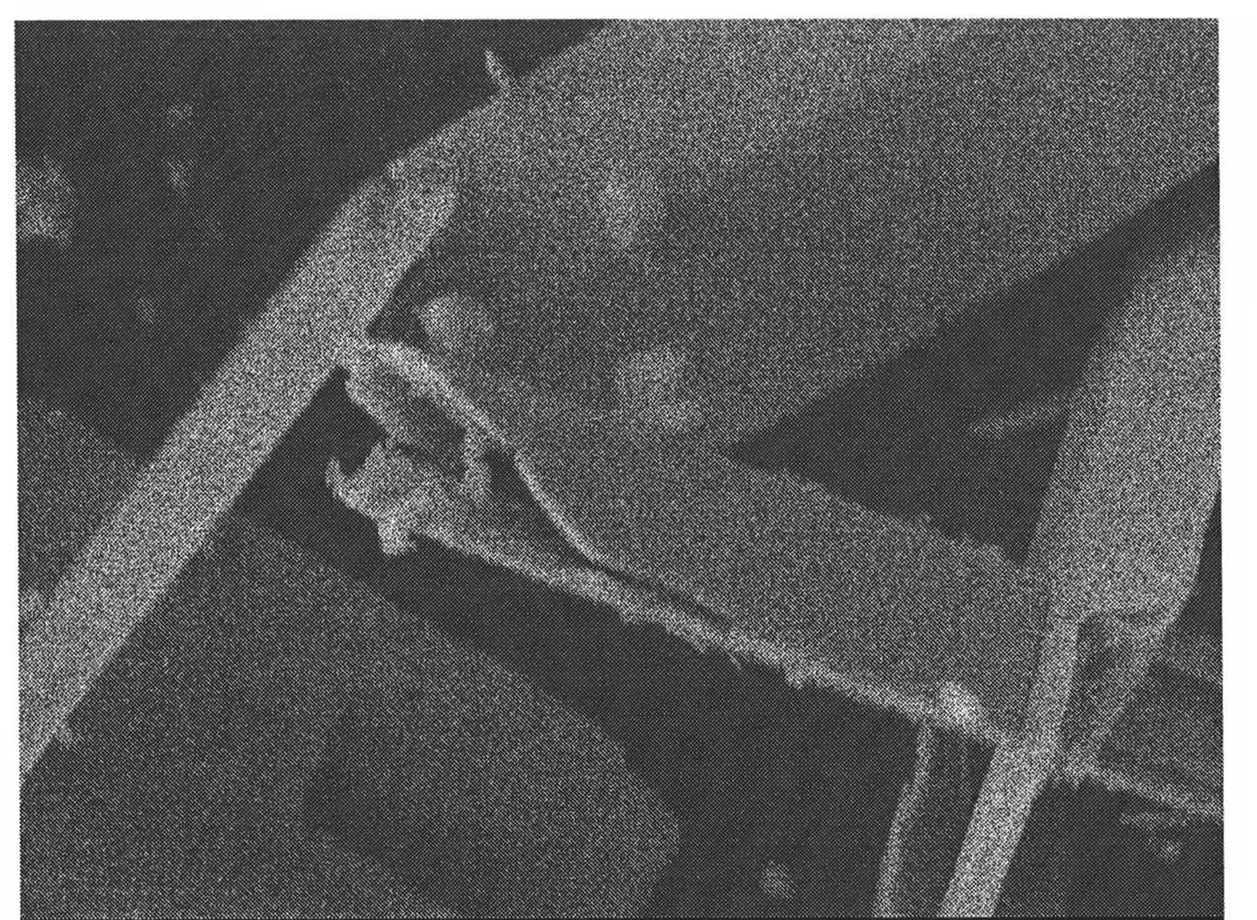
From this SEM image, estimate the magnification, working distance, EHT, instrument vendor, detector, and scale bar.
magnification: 4.5 K X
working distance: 11.5 mm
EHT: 10 kV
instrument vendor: JEOL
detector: LEI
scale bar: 1000 nm